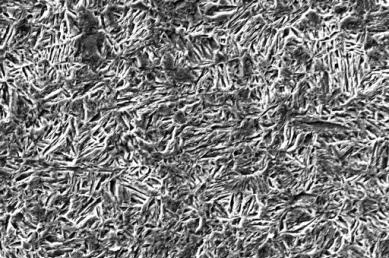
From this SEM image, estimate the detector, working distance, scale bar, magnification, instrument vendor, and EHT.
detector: SE1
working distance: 12 mm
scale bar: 10000 nm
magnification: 1 K X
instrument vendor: Zeiss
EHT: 20 kV